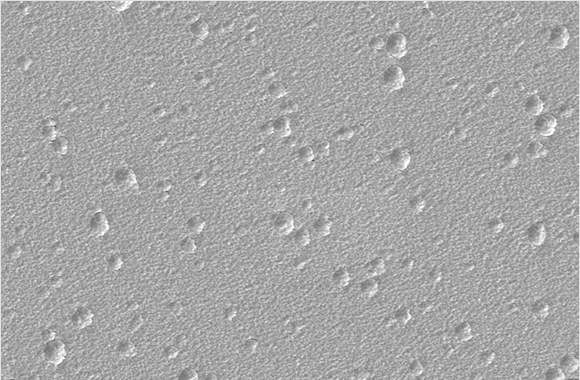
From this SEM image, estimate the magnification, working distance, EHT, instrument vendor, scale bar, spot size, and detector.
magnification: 5 K X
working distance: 5 mm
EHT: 5 kV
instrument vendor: FEI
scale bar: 10000 nm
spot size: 3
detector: ETD